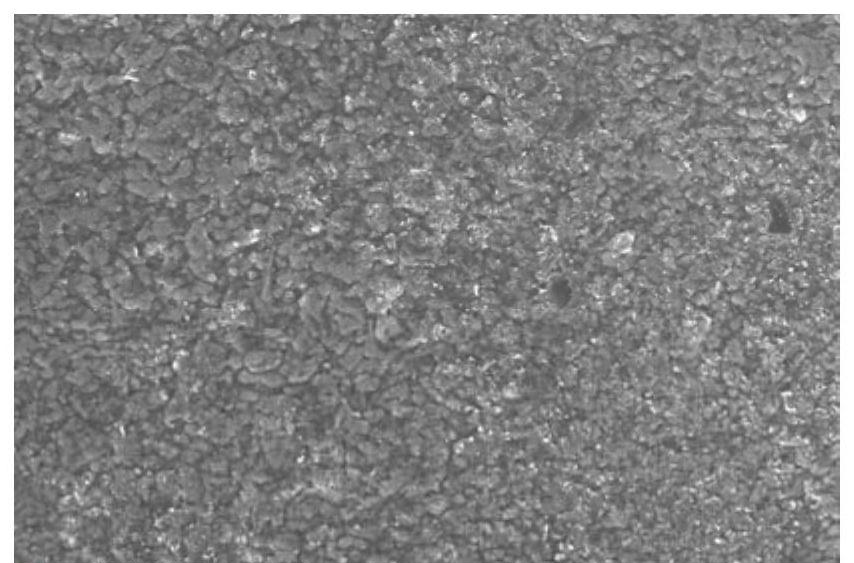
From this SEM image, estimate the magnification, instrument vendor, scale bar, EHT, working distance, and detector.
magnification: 2 K X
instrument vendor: Thermo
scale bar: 50000 nm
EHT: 10 kV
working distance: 10 mm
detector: ETD